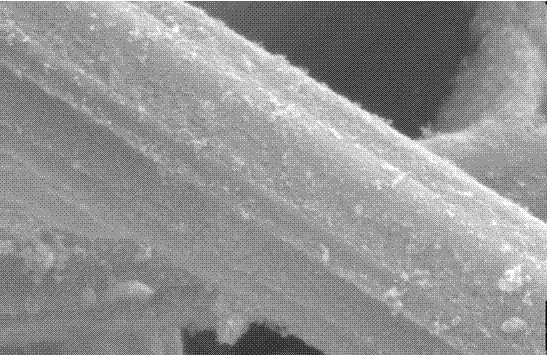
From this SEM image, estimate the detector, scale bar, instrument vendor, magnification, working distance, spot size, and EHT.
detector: SE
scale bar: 5000 nm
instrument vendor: FEI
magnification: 10 K X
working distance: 7 mm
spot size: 4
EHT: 25 kV